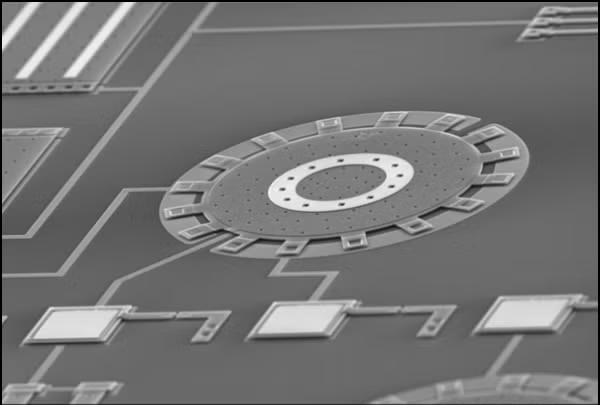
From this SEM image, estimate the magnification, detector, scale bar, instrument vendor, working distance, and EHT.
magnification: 0.2 K X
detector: SE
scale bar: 200000 nm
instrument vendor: JEOL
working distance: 31.7 mm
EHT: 25 kV